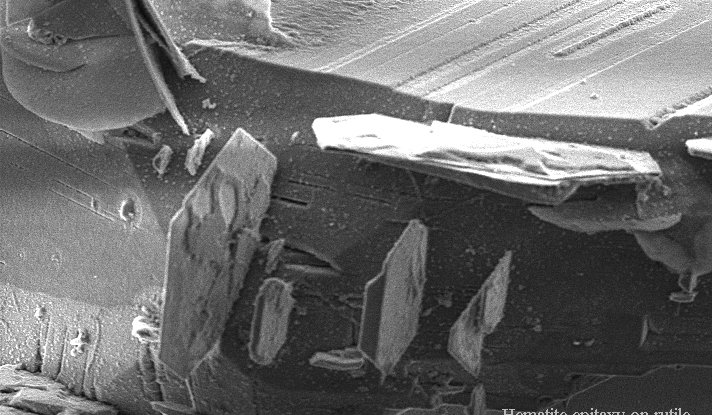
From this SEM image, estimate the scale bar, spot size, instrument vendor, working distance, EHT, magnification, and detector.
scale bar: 20000 nm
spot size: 3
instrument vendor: FEI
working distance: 6.4 mm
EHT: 10 kV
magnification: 0.8 K X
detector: SE